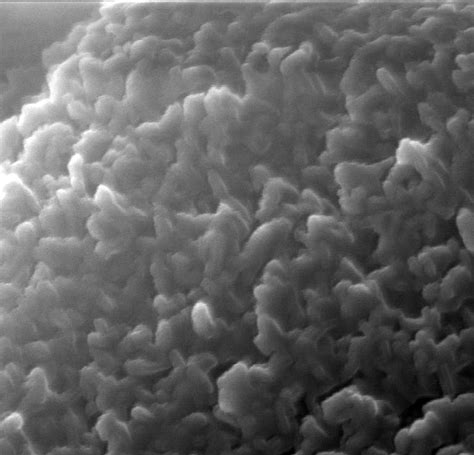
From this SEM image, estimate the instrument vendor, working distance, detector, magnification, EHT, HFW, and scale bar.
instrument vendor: Tescan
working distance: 6.802 mm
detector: SE Detector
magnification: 100 K X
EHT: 15 kV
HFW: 1.984 µm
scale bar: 500 nm